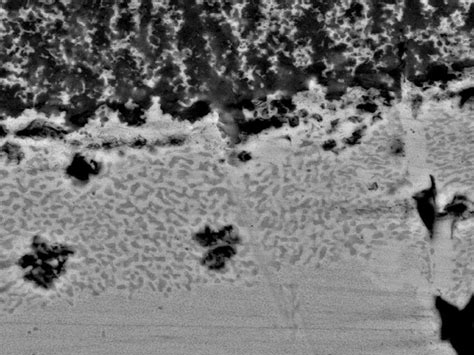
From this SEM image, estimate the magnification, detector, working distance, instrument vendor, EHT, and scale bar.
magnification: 10 K X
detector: A+B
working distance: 9.7 mm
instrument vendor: FEI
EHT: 25 kV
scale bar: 10000 nm